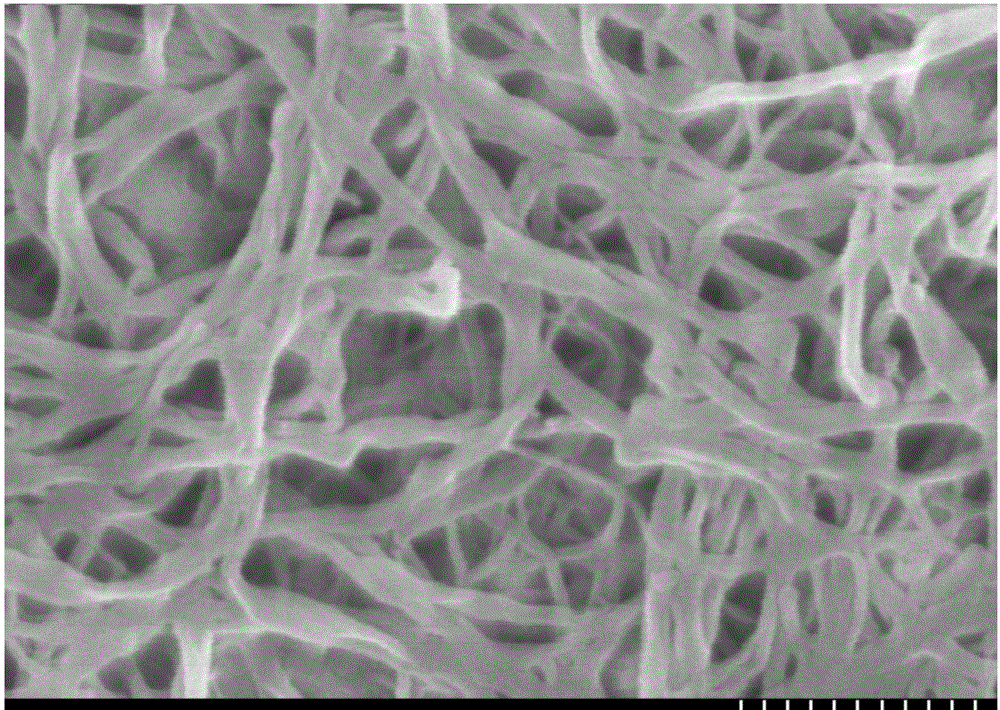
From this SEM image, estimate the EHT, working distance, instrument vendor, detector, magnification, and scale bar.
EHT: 15 kV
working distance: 12 mm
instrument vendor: Hitachi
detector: SE(M)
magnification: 60 K X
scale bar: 500 nm